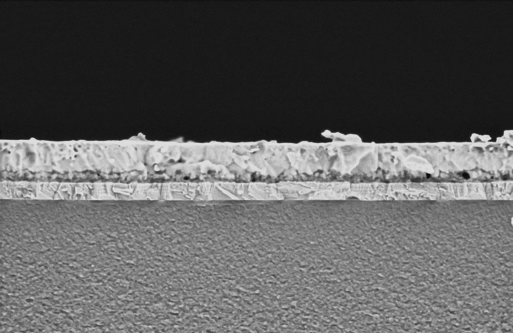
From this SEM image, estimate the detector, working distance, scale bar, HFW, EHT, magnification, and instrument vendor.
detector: T1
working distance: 3.5 mm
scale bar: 1000 nm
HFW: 4.23 µm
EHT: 1 kV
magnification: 30 K X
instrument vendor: Thermo